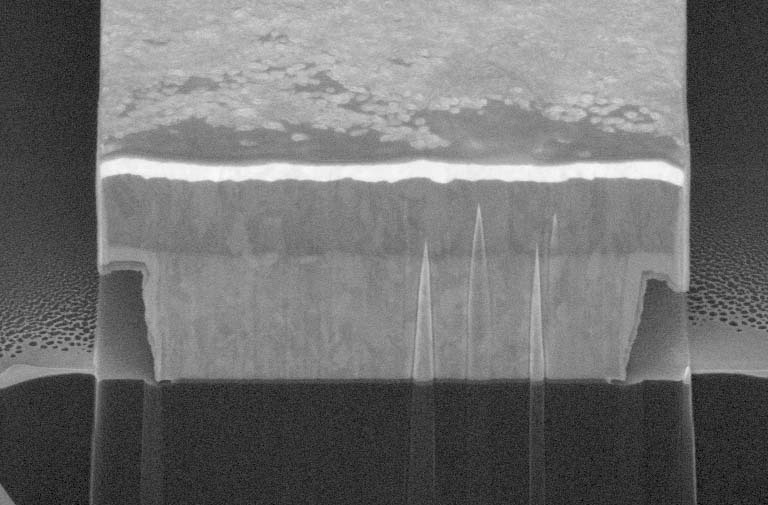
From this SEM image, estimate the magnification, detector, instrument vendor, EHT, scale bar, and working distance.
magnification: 8 K X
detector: TLD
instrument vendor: FEI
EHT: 5 kV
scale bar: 3000 nm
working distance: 4.3 mm